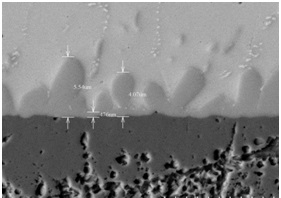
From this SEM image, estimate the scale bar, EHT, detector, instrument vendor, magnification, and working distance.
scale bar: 10000 nm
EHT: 15 kV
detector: BSE3D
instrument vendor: Hitachi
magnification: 5 K X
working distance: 8.9 mm